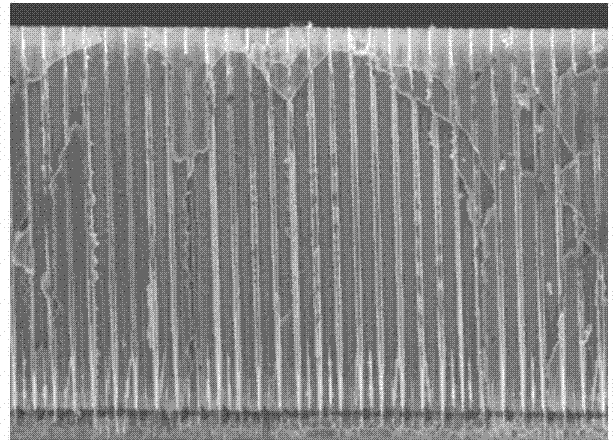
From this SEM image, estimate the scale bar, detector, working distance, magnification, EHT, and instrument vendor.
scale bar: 50000 nm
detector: SE(U)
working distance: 15.5 mm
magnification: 0.7 K X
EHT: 10 kV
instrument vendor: Hitachi